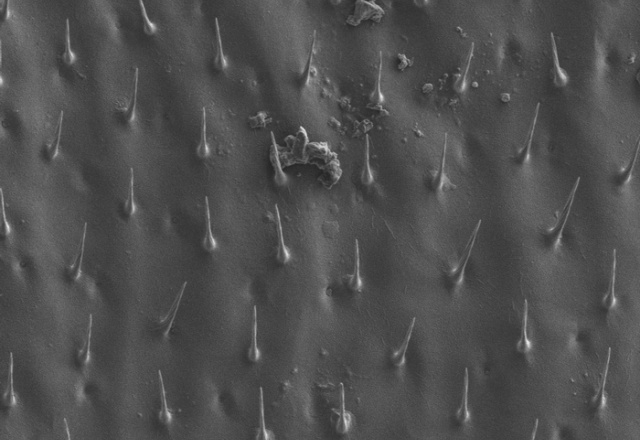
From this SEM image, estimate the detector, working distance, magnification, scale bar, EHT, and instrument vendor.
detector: LM(L)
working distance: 7.8 mm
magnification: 0.7 K X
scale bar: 50000 nm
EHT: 5 kV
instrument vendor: Hitachi